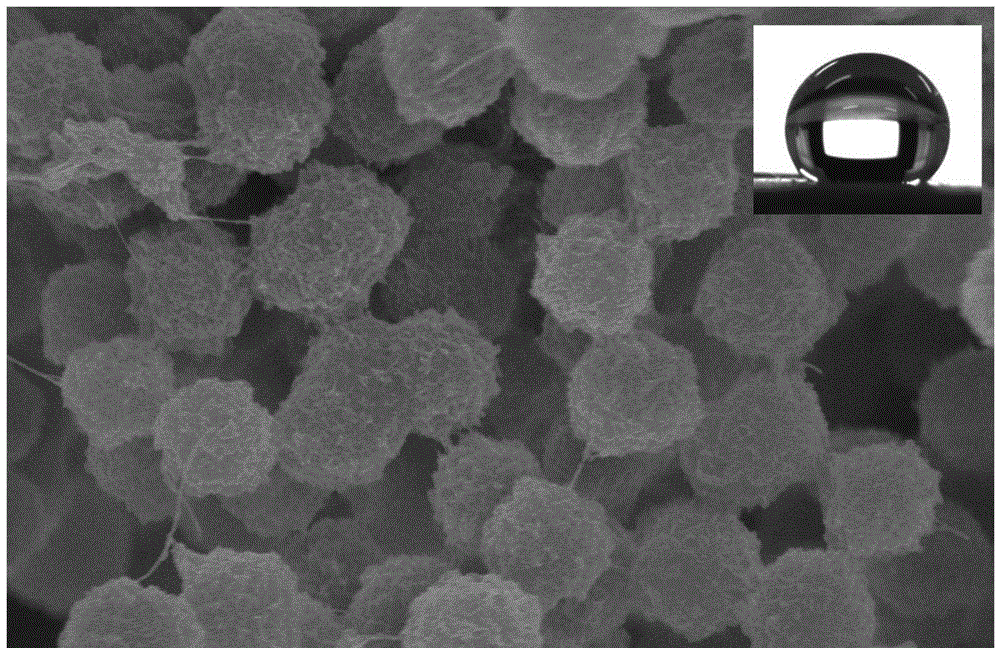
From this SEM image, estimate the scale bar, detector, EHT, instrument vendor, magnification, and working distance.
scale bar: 5000 nm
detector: SE(M)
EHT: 10 kV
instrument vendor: Hitachi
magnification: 8 K X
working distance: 6.3 mm